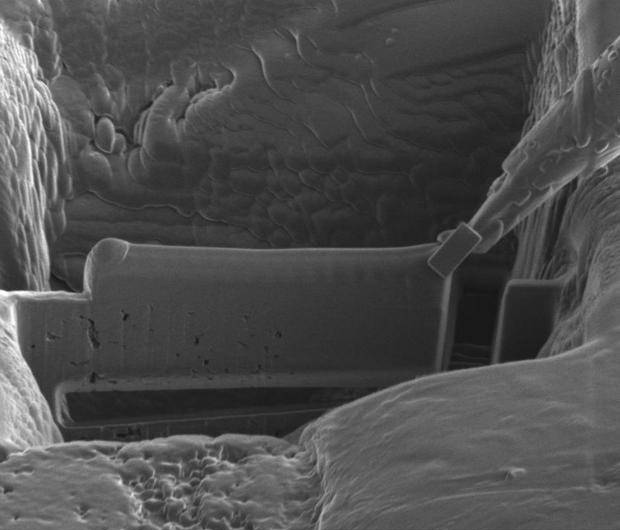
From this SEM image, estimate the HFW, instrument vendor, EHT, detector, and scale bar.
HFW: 25.6 µm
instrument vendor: FEI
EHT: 30 kV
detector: ICE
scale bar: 5000 nm